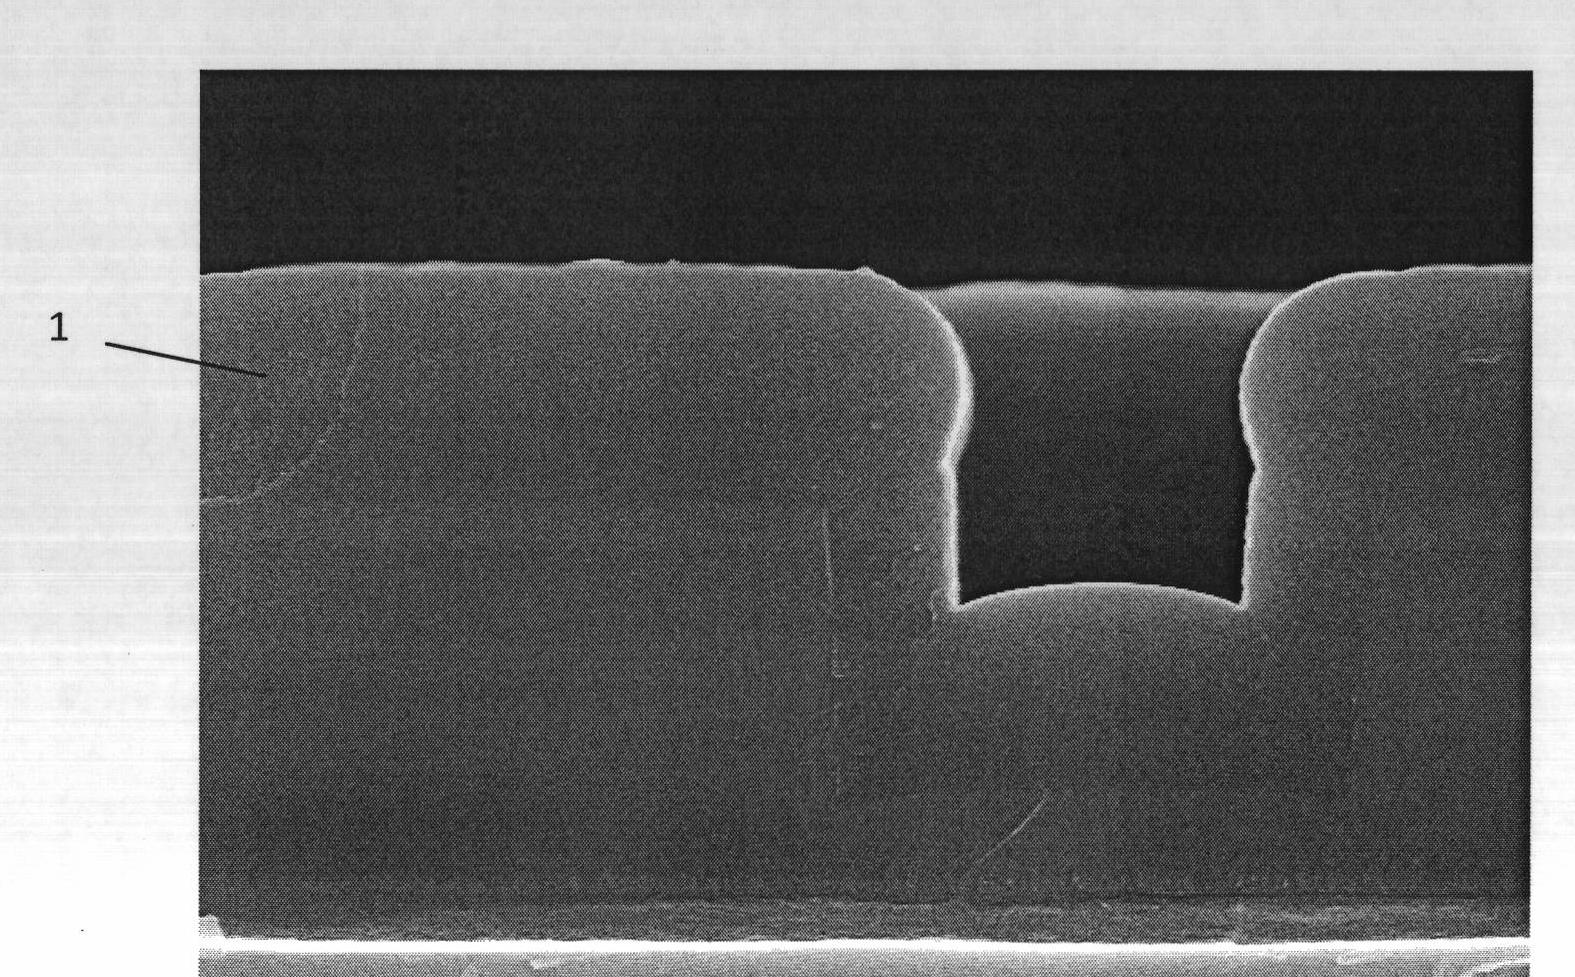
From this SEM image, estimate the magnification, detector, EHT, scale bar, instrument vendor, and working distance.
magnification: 30 K X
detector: SE(U)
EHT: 15 kV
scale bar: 1000 nm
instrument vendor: Hitachi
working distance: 6.1 mm